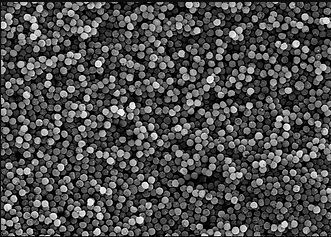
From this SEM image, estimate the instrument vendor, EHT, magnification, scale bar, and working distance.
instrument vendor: Hitachi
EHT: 15 kV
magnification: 2 K X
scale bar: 20000 nm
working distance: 15.7 mm